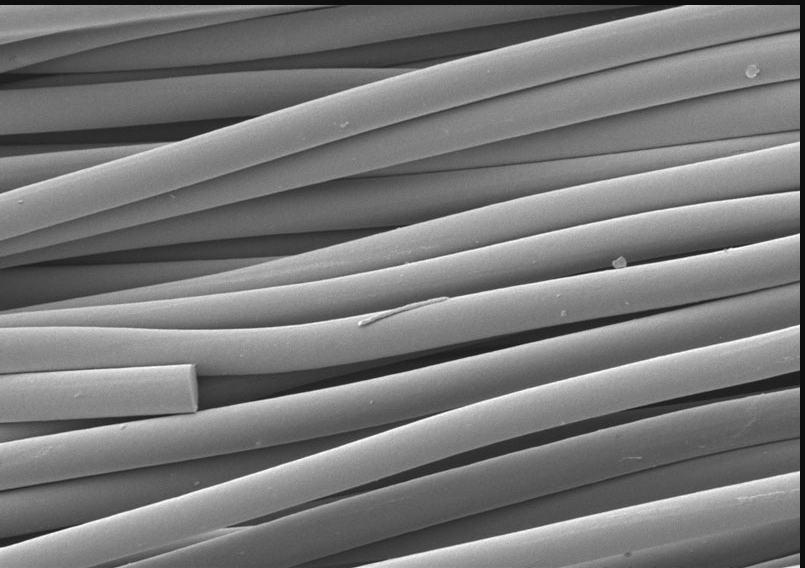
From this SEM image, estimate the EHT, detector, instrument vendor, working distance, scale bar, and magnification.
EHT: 3 kV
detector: SE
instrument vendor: Hitachi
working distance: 10.7 mm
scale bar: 20000 nm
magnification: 2 K X